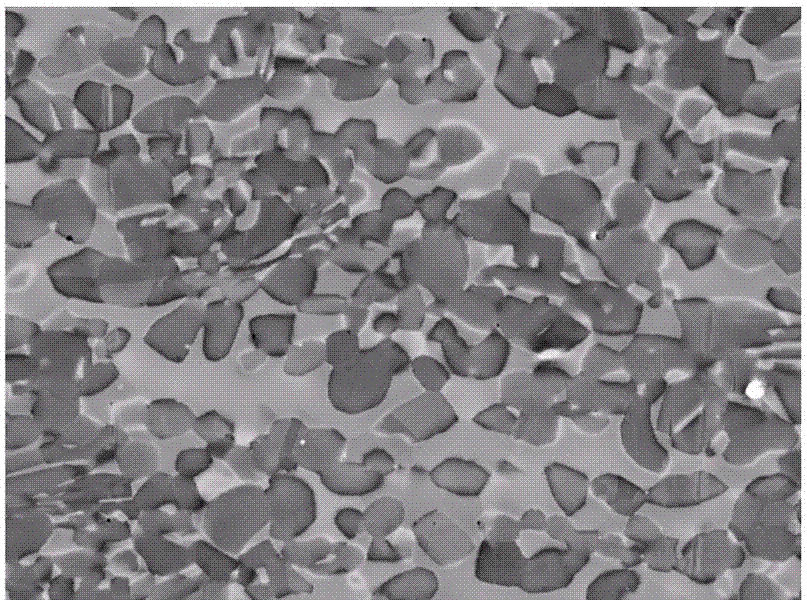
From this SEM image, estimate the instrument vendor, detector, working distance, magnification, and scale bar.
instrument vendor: Tescan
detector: BSE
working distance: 12.07 mm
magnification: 4 K X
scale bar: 20000 nm